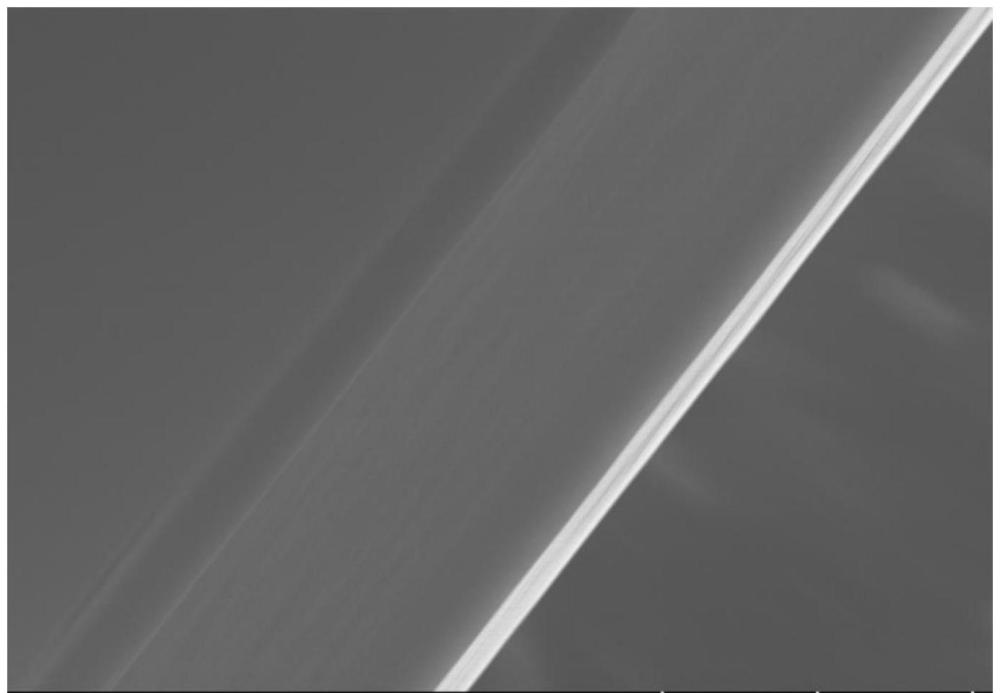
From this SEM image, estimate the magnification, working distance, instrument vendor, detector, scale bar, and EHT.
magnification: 4 K X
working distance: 9.7 mm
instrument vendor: Hitachi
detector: SE(UL)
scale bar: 10000 nm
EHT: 10 kV